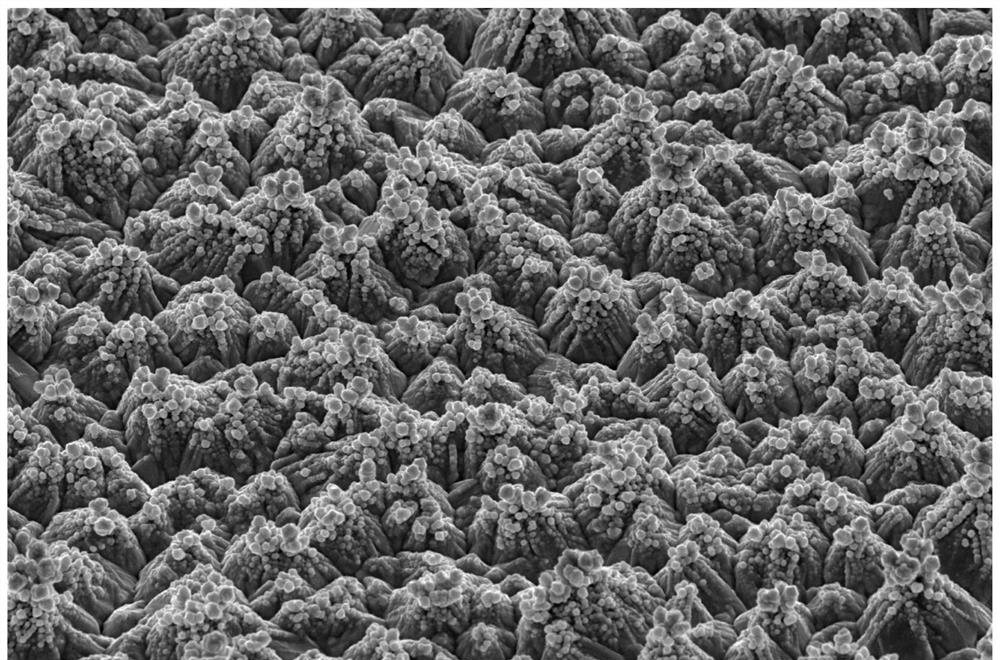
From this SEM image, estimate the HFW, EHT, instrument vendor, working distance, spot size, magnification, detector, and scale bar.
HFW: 82.9 µm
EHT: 20 kV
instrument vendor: FEI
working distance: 16.3 mm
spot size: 3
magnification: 5 K X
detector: ETD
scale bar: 30000 nm